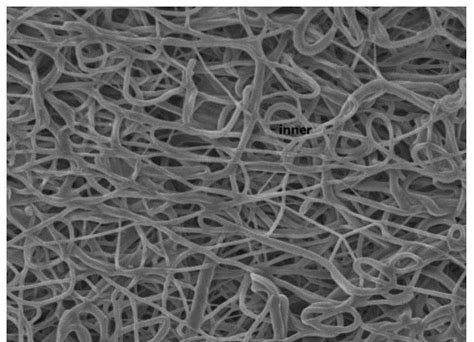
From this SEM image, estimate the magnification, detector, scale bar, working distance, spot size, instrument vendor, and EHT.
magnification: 1 K X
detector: SED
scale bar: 10000 nm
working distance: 13.8 mm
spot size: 30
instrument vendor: JEOL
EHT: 10 kV